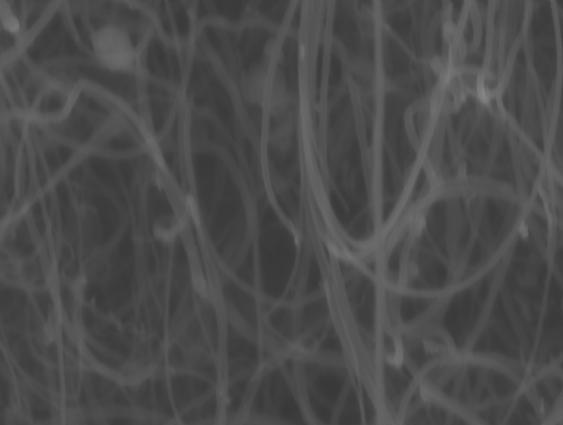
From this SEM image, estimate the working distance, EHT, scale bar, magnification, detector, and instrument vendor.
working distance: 5 mm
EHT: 5 kV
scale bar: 200 nm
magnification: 40 K X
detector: InLens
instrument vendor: Zeiss